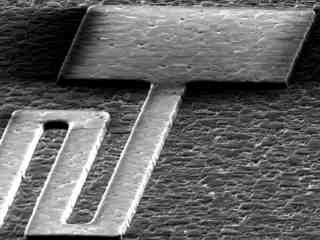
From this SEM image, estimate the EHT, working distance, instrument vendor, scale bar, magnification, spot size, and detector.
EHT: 17 kV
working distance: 12.4 mm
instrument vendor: FEI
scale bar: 20000 nm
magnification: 0.8 K X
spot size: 3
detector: SE